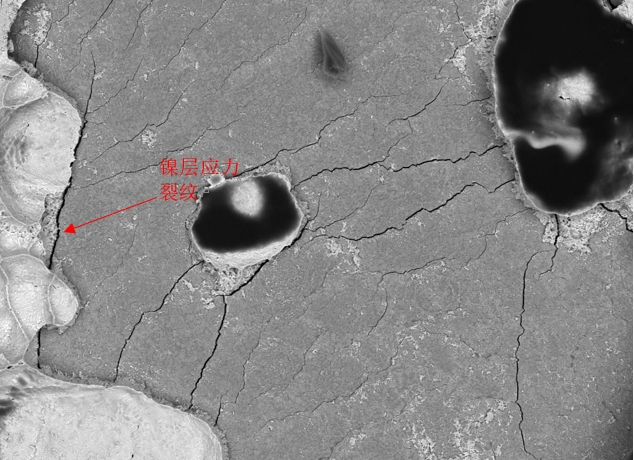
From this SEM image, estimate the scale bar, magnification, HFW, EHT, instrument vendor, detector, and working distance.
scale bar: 50000 nm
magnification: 0.907 K X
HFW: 305 µm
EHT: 20 kV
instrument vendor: Tescan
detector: BSE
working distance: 14.3 mm